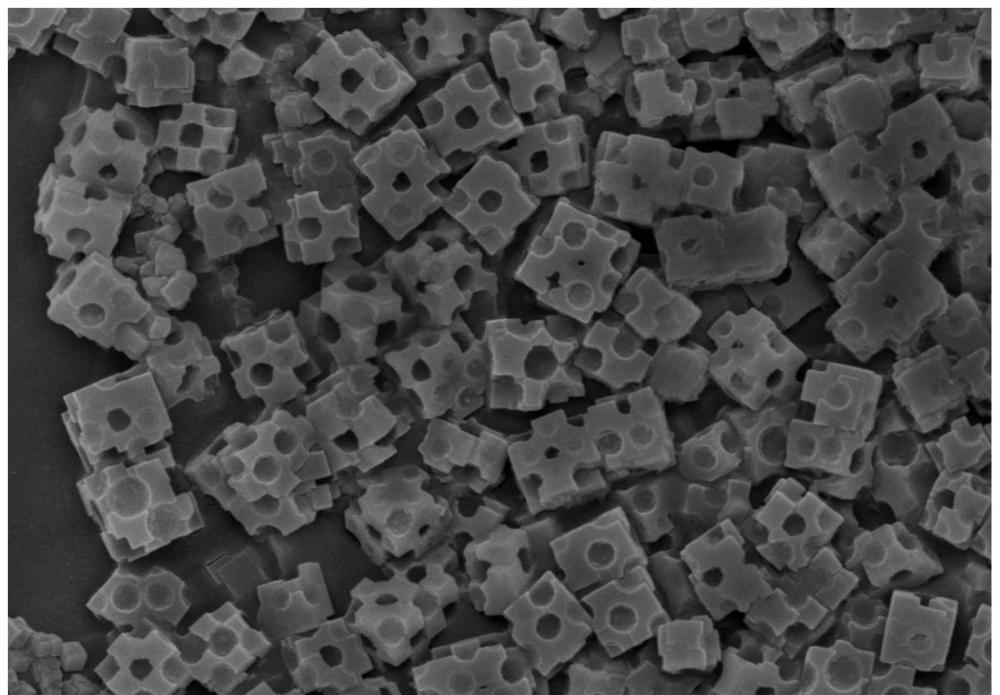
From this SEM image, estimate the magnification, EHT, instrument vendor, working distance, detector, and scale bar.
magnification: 20 K X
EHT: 5 kV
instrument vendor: Hitachi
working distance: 8.2 mm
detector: SE(U)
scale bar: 2000 nm